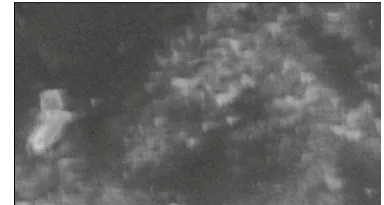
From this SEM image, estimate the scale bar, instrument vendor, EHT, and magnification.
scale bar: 5000 nm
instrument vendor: JEOL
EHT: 15 kV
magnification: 5 K X